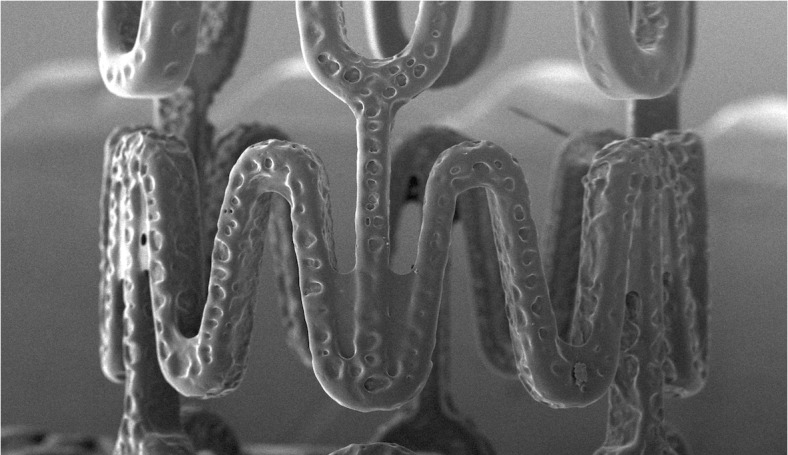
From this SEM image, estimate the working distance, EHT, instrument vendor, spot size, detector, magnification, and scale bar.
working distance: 5.5 mm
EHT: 10 kV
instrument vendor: FEI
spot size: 5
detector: SE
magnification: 0.12 K X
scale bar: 500000 nm